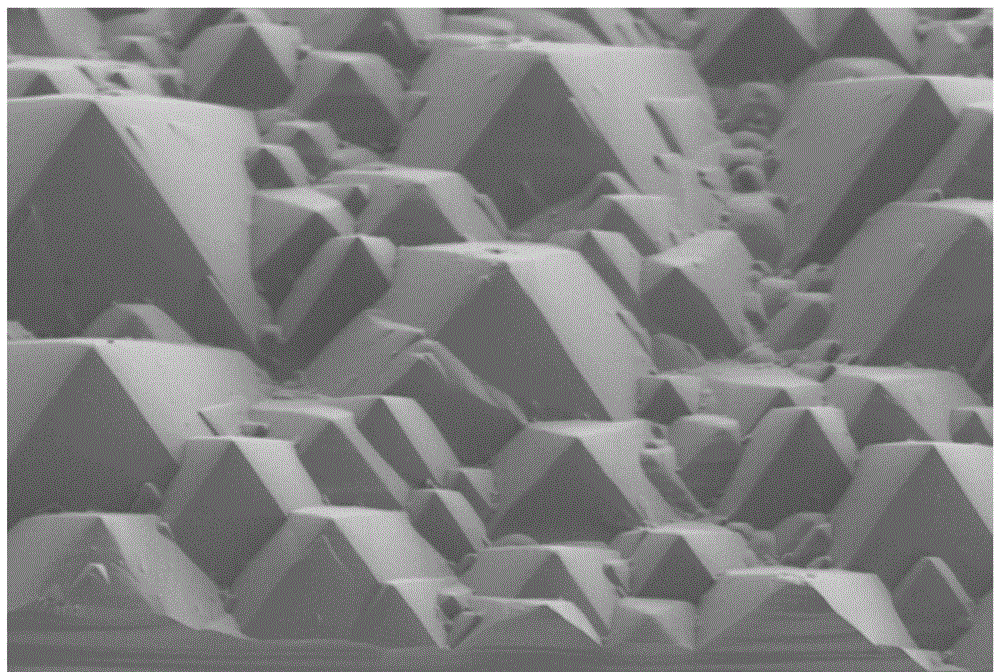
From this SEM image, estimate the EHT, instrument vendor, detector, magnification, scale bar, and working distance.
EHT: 1 kV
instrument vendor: Hitachi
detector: SE(L)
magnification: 3 K X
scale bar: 10000 nm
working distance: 11.6 mm